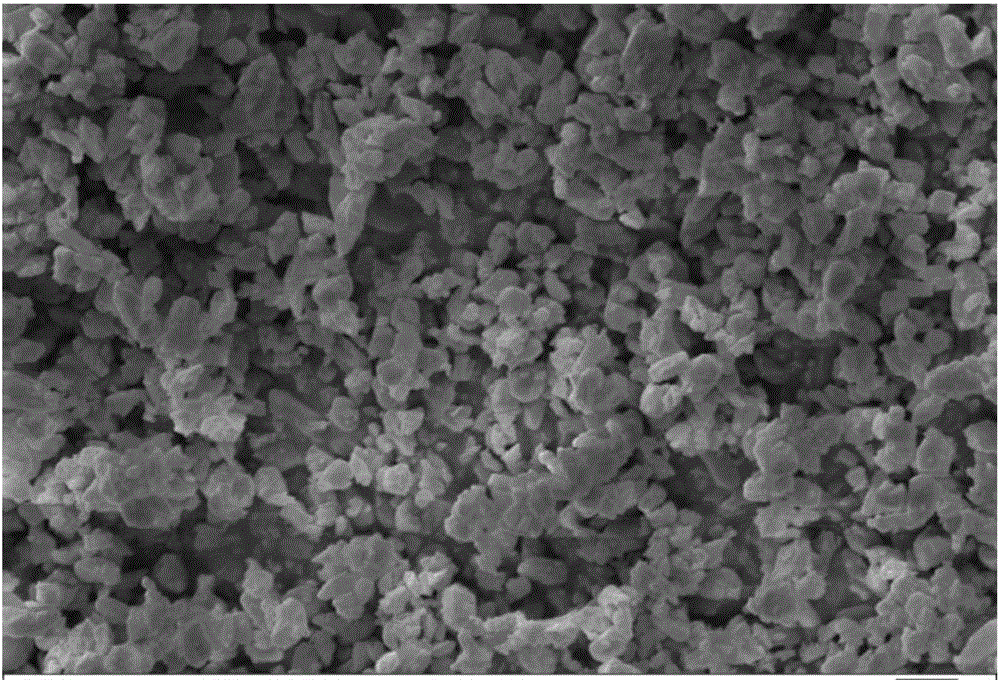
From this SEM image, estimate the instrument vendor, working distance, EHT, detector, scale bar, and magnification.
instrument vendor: Zeiss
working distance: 8.5 mm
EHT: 10 kV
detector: SE1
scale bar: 1000 nm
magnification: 5 K X